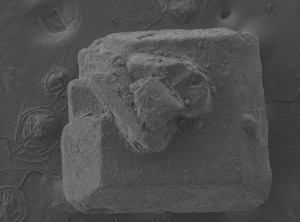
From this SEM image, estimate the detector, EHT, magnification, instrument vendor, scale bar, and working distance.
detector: LEI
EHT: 5 kV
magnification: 0.085 K X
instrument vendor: JEOL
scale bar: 100000 nm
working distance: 8 mm